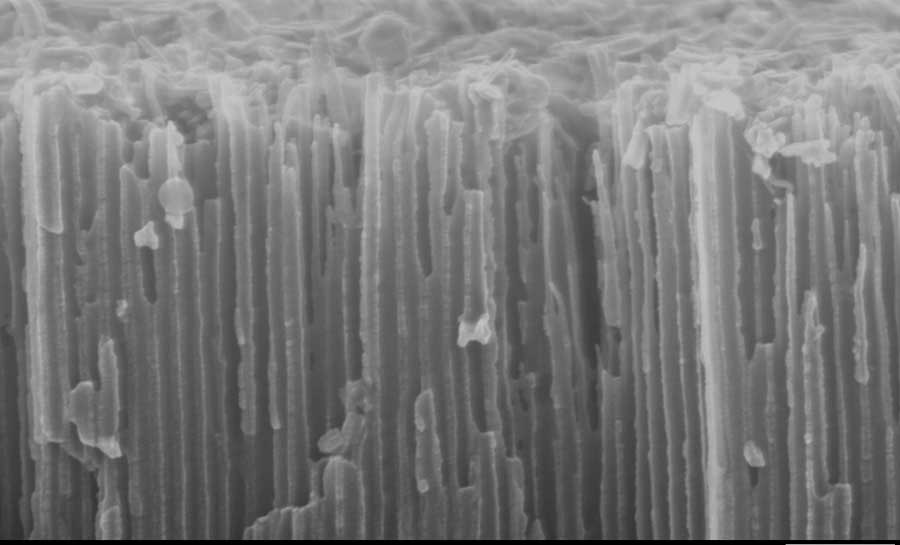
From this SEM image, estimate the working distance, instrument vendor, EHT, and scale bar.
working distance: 3 mm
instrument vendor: Zeiss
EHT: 10 kV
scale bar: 100 nm